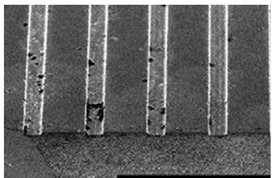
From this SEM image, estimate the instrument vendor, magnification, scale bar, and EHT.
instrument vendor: JEOL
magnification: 0.1 K X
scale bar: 200000 nm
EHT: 25 kV